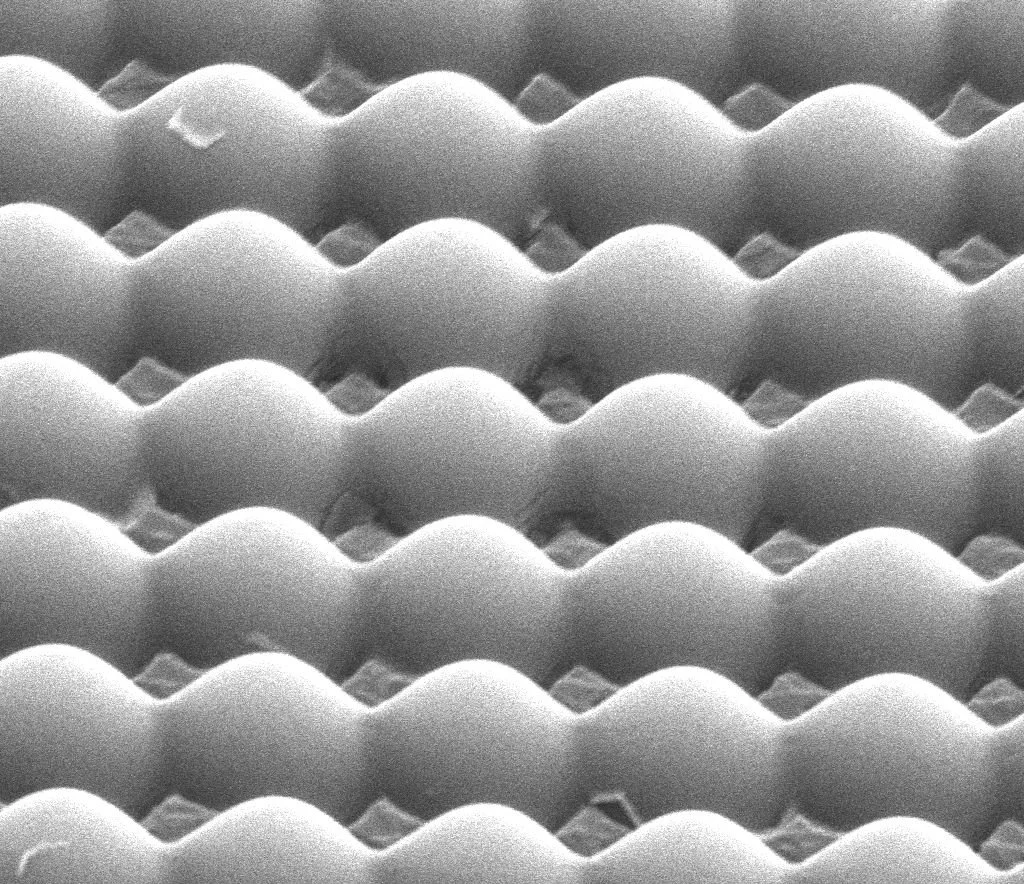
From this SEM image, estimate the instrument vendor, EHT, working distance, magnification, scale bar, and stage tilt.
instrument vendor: FEI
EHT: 10 kV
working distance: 11.1 mm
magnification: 15 K X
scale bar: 4000 nm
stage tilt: -0°